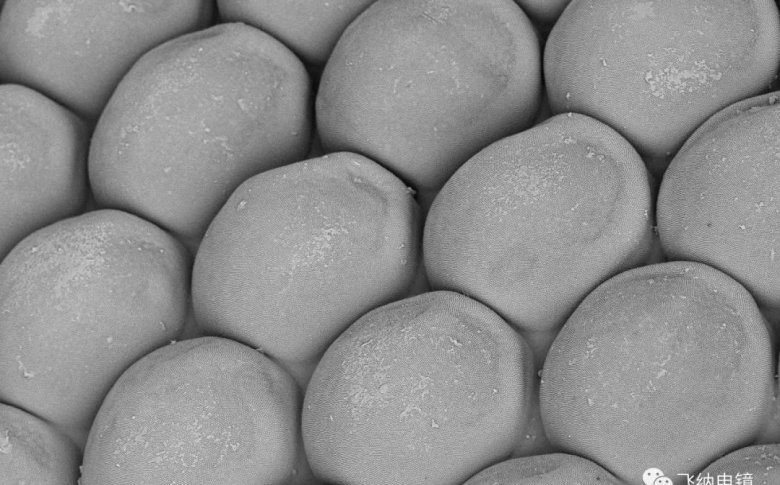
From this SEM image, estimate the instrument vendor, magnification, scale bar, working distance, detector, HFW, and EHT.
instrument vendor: other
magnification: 2 K X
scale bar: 20000 nm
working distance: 8.545 mm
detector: BSD Full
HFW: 63.5 µm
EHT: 5 kV